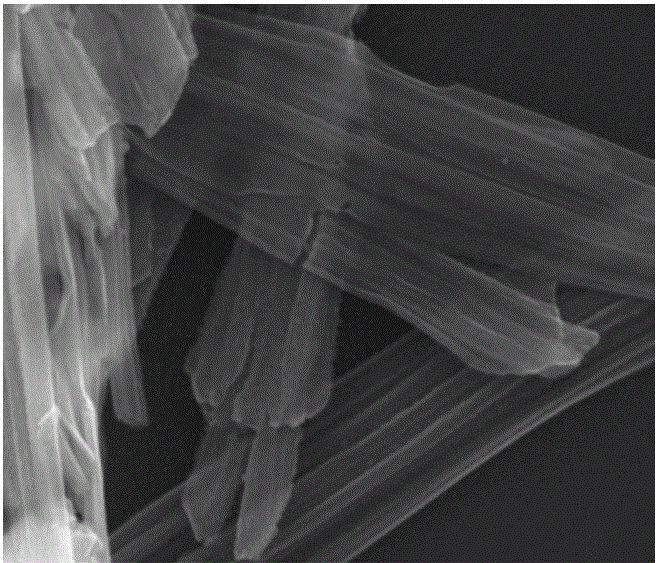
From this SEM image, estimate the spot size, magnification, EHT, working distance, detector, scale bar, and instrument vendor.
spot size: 3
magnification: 80 K X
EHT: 20 kV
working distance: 9.6 mm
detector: ETD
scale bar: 1000 nm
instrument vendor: FEI